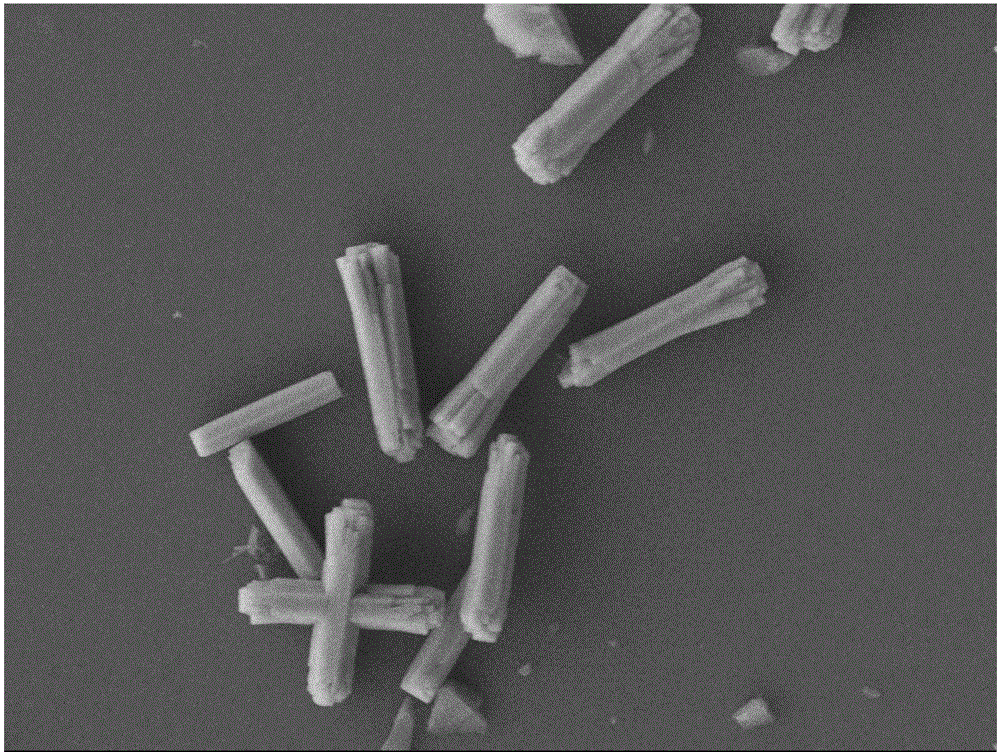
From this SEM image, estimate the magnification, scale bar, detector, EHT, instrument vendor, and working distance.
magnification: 5 K X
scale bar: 1000 nm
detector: LEI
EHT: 8 kV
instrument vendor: JEOL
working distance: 7.3 mm